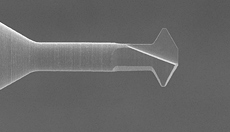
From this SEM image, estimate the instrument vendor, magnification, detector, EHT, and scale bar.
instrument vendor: JEOL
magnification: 0.5 K X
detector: SEI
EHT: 10 kV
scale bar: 50000 nm